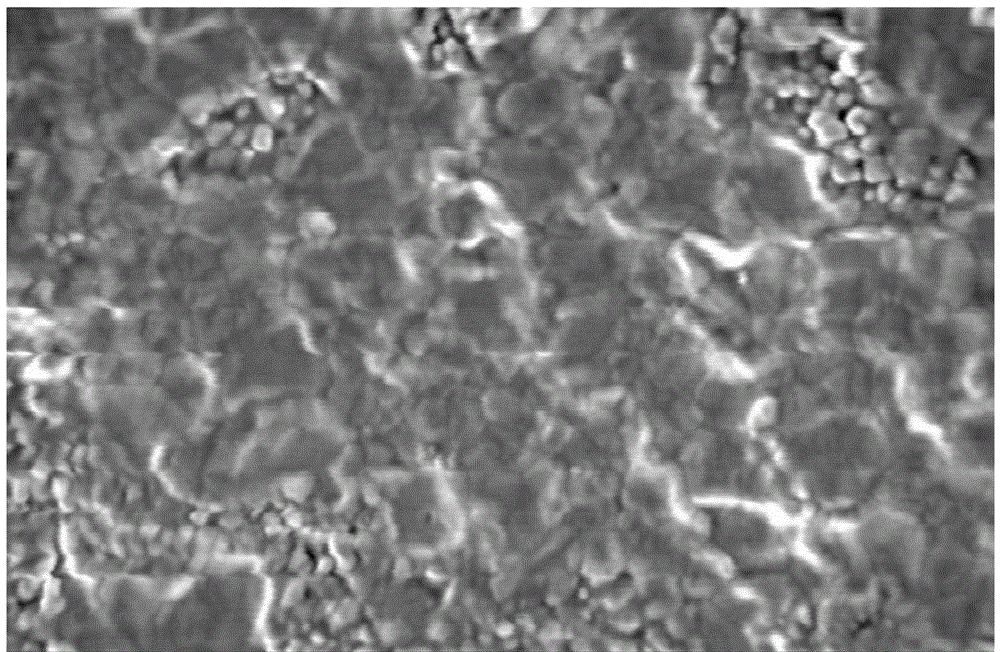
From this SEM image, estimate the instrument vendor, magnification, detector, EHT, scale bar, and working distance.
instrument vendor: Hitachi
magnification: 50 K X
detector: SE(M)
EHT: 1.5 kV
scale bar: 1000 nm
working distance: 4.6 mm